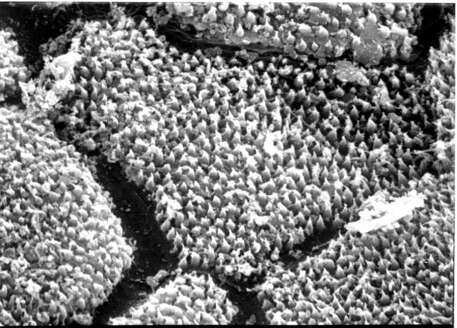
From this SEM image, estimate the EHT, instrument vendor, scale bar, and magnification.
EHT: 20 kV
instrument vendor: JEOL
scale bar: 10000 nm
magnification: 5 K X